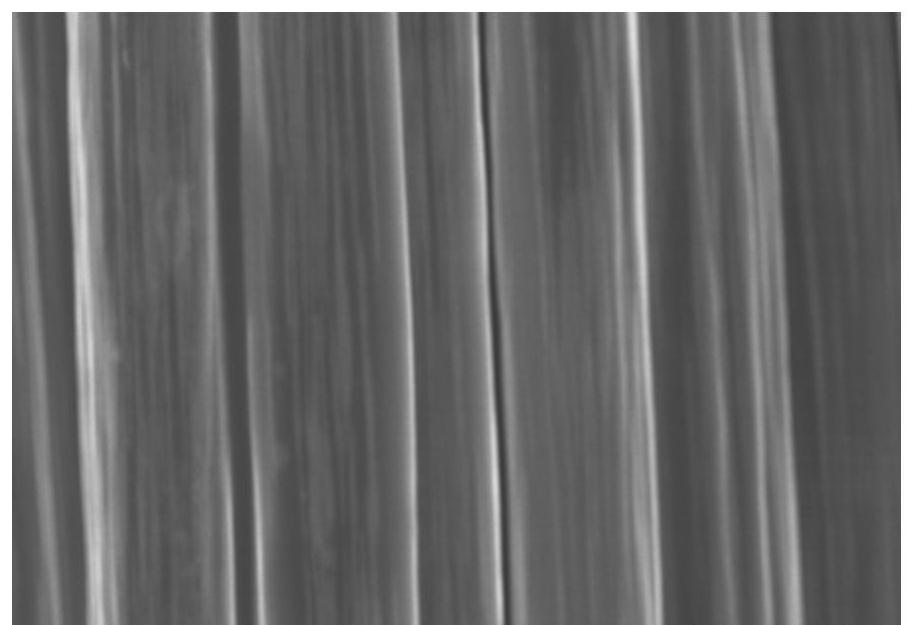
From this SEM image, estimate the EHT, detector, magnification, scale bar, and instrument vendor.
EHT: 8 kV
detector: SE(M)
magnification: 3 K X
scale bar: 10000 nm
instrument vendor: Hitachi